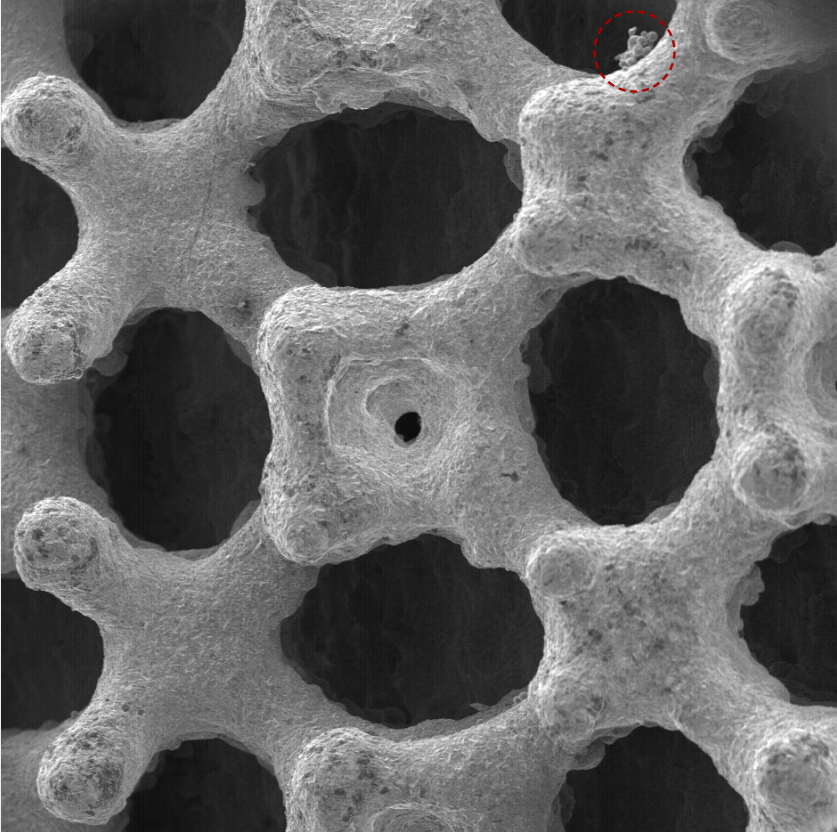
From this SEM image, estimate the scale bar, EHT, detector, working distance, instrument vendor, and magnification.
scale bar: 500000 nm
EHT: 10 kV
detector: SED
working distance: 2.45 mm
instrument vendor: other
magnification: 0.109 K X